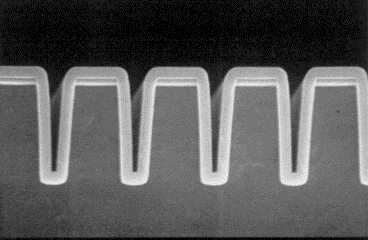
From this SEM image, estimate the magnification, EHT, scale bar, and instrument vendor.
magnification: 9.15 K X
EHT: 30 kV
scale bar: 10000 nm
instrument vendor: other